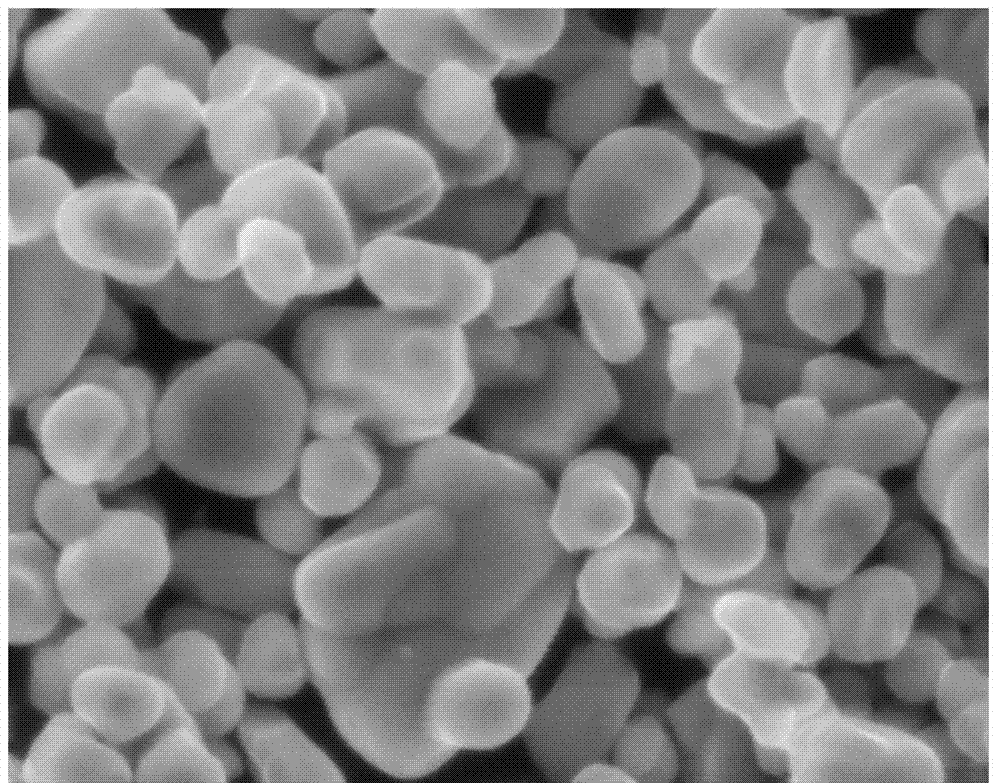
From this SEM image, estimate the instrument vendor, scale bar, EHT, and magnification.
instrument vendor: JEOL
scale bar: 5000 nm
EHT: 15 kV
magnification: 5 K X